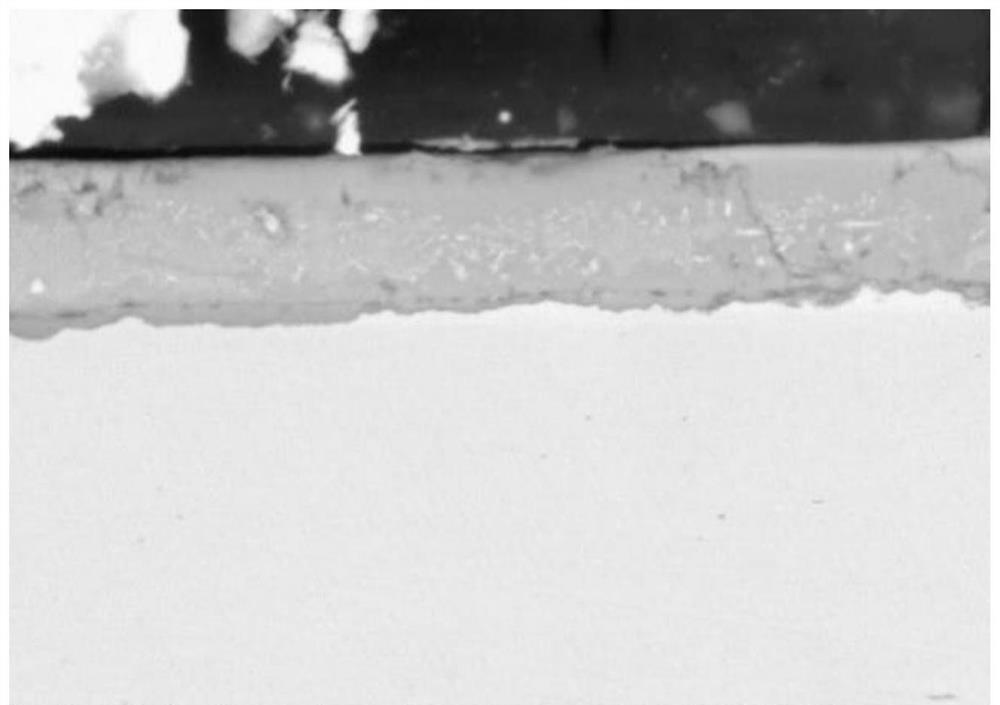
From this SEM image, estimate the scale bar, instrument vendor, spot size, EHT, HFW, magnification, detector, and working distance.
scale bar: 5000 nm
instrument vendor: FEI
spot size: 4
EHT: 20 kV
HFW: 59.7 µm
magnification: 5 K X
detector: BSED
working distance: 10.8 mm